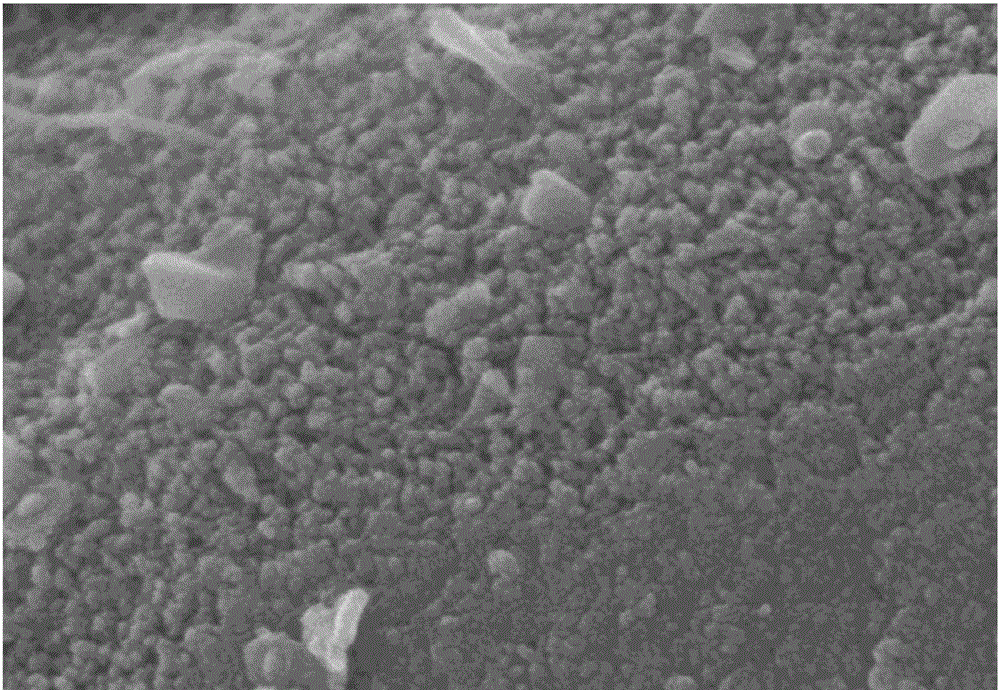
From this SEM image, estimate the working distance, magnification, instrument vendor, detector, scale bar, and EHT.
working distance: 8.4 mm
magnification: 100 K X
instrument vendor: Hitachi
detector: SE(U)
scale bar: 500 nm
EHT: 5 kV